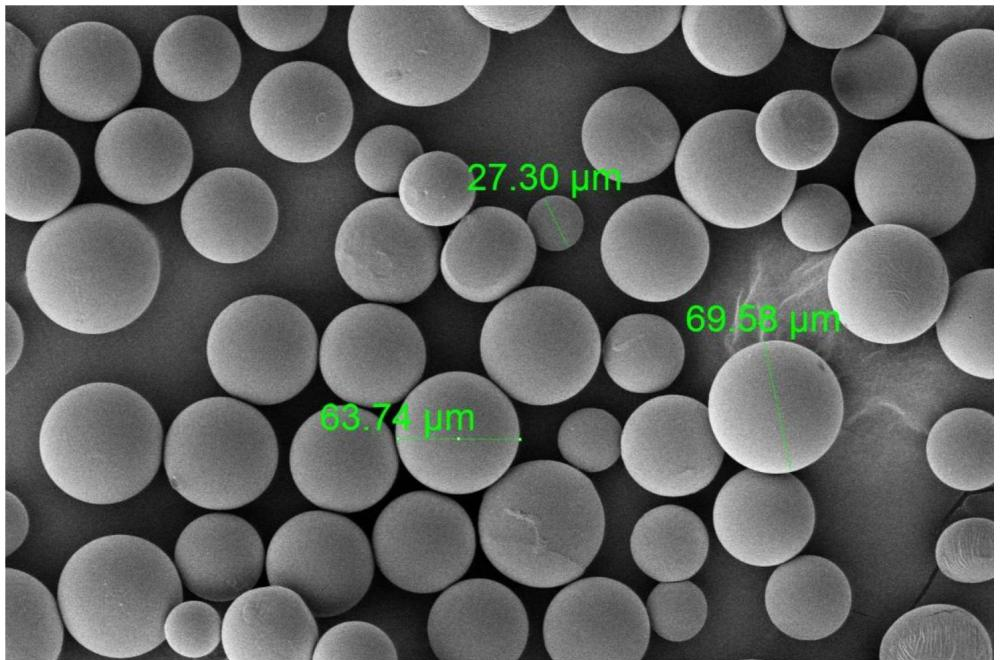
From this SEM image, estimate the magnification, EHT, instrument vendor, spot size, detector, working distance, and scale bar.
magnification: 0.8 K X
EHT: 5 kV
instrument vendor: FEI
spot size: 3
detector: ETD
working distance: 5.3 mm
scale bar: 200000 nm